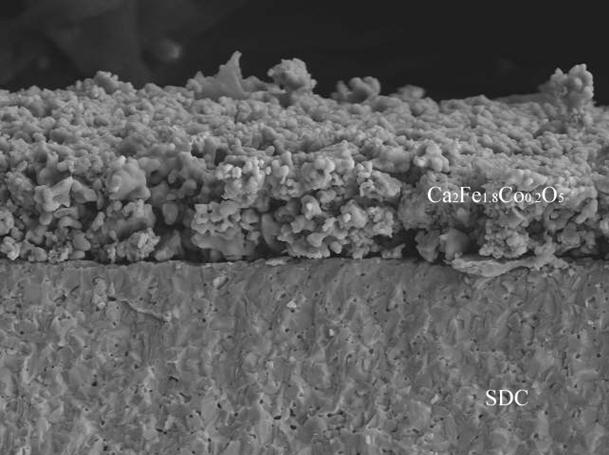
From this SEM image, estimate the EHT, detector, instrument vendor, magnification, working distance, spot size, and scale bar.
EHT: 20 kV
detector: SE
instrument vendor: FEI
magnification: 1 K X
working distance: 14.9 mm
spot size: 5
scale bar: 20000 nm